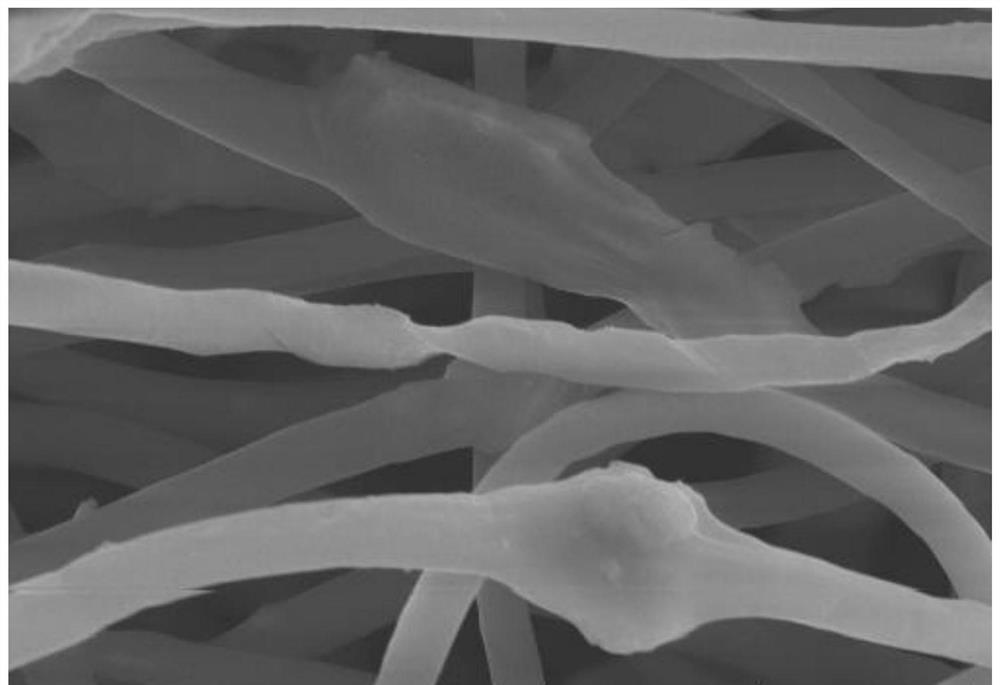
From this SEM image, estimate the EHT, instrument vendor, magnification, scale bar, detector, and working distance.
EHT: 10 kV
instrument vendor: Hitachi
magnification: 11 K X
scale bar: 5000 nm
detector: SE(UL)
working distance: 13.1 mm